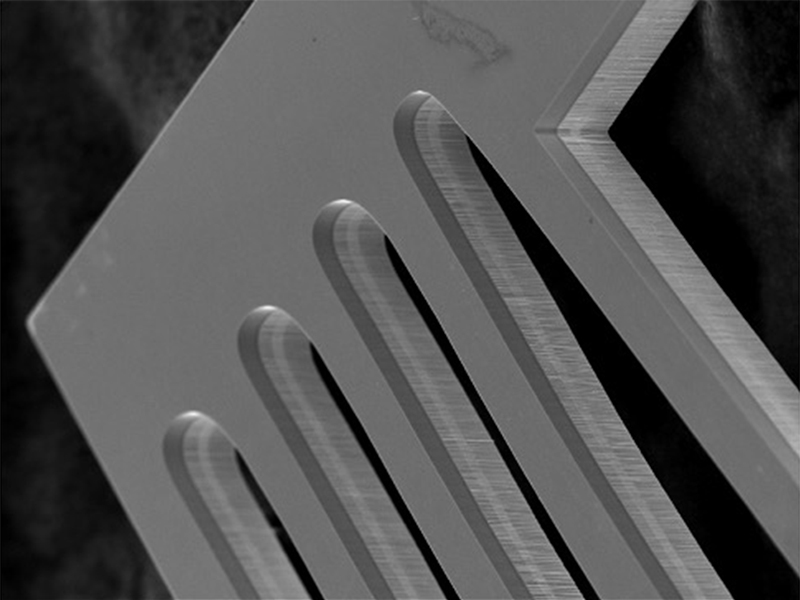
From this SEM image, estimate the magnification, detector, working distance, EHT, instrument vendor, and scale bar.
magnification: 0.8 K X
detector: SEI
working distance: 10.6 mm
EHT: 20 kV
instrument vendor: JEOL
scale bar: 50000 nm